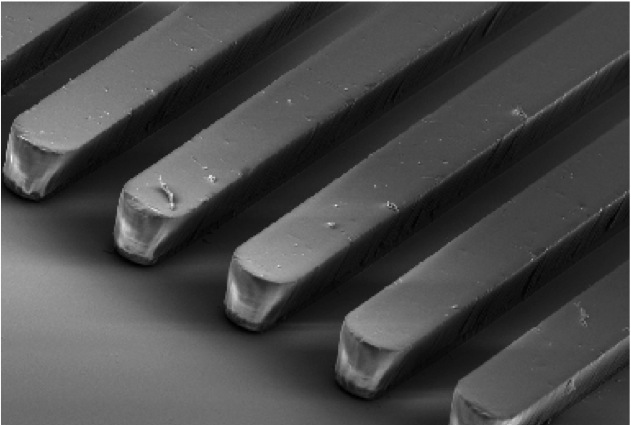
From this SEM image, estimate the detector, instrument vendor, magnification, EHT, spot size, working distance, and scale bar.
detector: SEI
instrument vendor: JEOL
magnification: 0.2 K X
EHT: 10 kV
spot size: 50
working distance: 32 mm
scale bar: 100000 nm